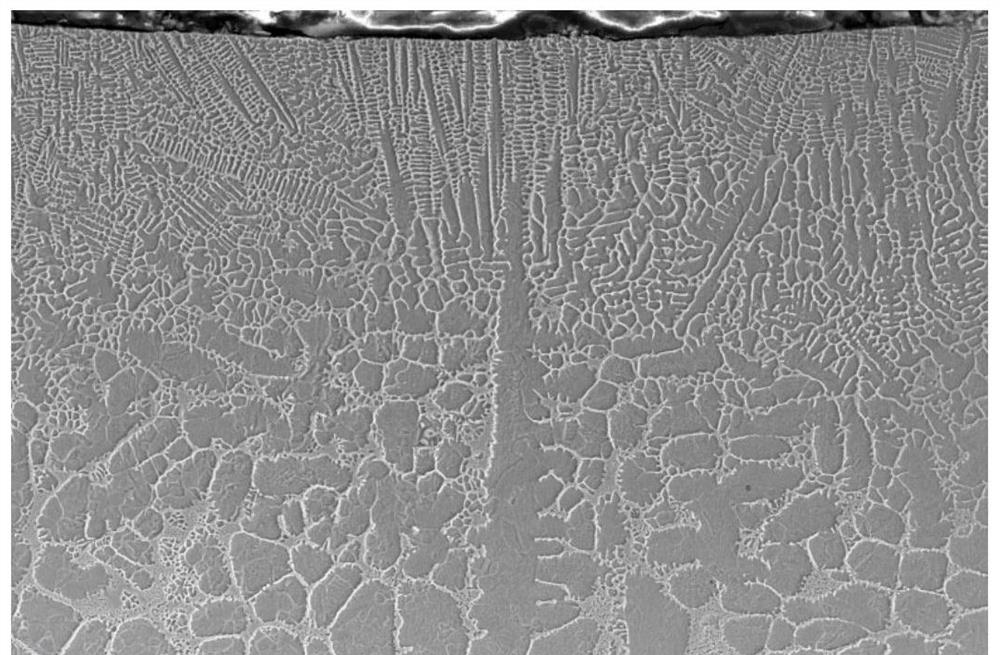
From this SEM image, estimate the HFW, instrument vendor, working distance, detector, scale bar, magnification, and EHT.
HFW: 207 µm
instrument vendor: Thermo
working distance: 9.6 mm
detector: ETD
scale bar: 50000 nm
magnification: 2 K X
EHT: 15 kV